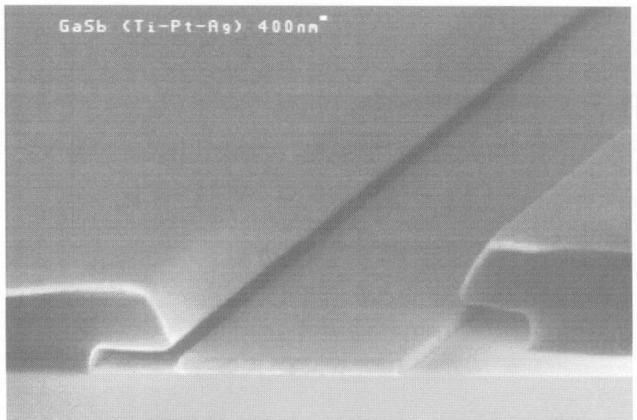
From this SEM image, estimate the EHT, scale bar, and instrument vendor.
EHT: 20 kV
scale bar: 3000 nm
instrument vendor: JEOL